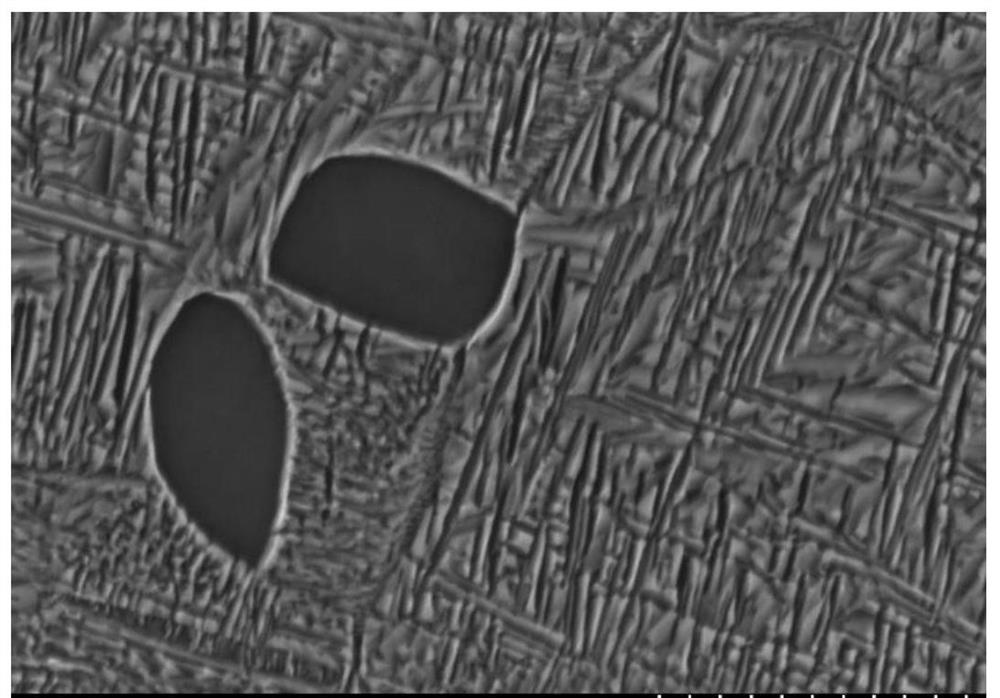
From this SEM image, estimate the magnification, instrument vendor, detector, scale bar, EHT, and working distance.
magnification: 20 K X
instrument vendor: Hitachi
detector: SE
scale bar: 2000 nm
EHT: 15 kV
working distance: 20.1 mm